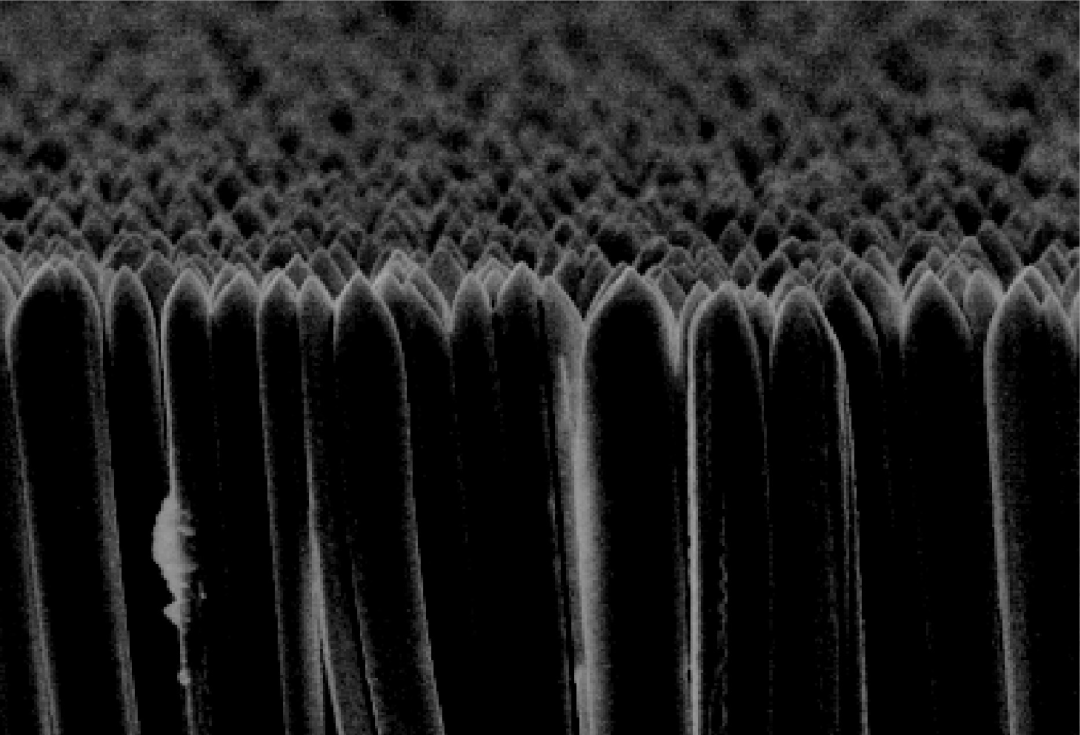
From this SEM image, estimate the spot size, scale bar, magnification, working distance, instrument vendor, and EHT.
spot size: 10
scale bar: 10000 nm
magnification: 3 K X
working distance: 5 mm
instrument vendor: JEOL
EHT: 20 kV